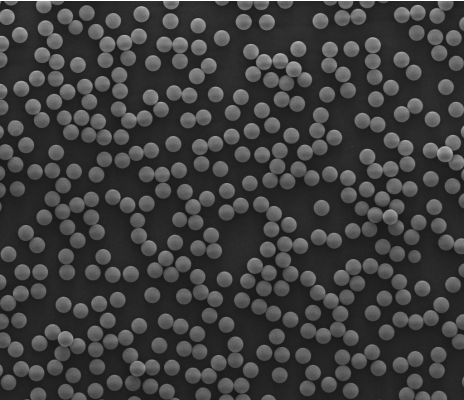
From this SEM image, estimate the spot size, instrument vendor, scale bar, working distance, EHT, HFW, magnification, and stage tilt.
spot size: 3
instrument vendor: FEI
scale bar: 50000 nm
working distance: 8.9 mm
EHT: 20 kV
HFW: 186 µm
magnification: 1.6 K X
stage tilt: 0°